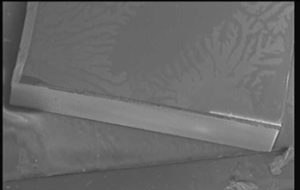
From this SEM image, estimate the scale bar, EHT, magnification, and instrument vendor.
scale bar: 500000 nm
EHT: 3 kV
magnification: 0.037 K X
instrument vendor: Hitachi